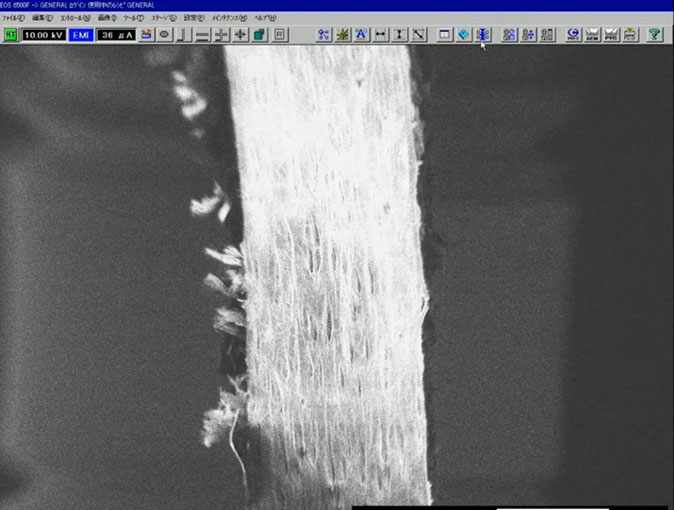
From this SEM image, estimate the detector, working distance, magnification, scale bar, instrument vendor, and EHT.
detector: SEI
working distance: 20 mm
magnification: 0.02 K X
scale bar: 1000000 nm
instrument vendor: JEOL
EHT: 10 kV